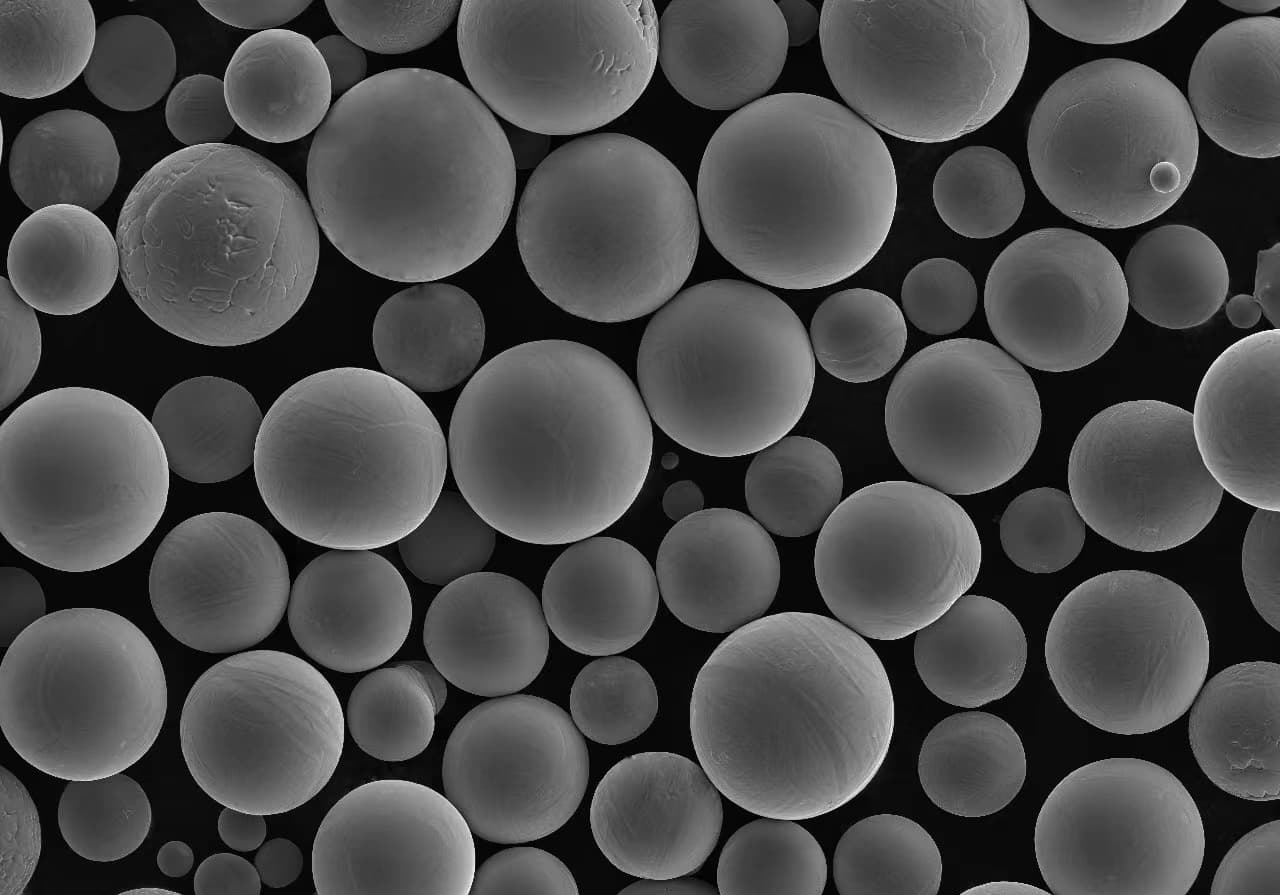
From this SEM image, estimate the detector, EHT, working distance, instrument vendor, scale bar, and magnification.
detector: SE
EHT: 15 kV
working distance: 7.6 mm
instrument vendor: Hitachi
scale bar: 200000 nm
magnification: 0.2 K X